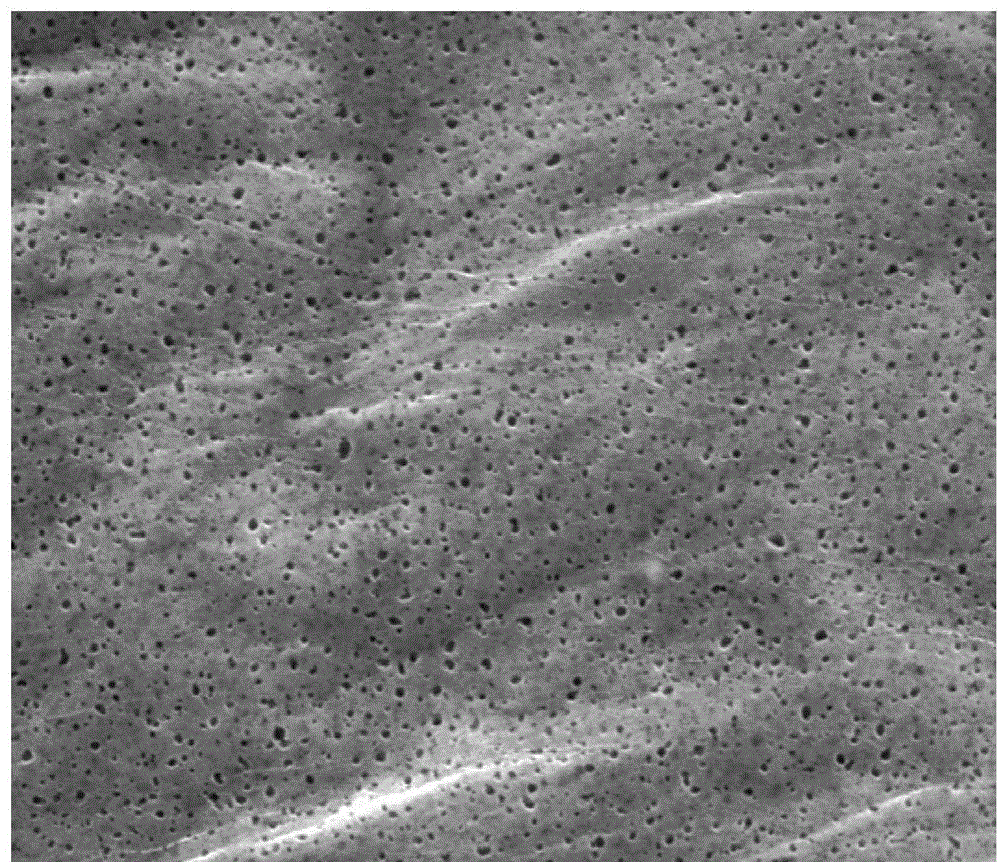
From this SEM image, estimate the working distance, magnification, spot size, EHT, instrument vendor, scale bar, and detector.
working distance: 10 mm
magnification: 50 K X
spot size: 3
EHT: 5 kV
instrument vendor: FEI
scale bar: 3000 nm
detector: ETD